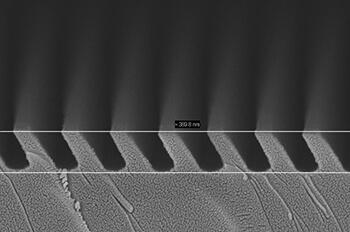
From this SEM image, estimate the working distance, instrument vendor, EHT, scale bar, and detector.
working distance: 4 mm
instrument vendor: Zeiss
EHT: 5 kV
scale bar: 200 nm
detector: InLens